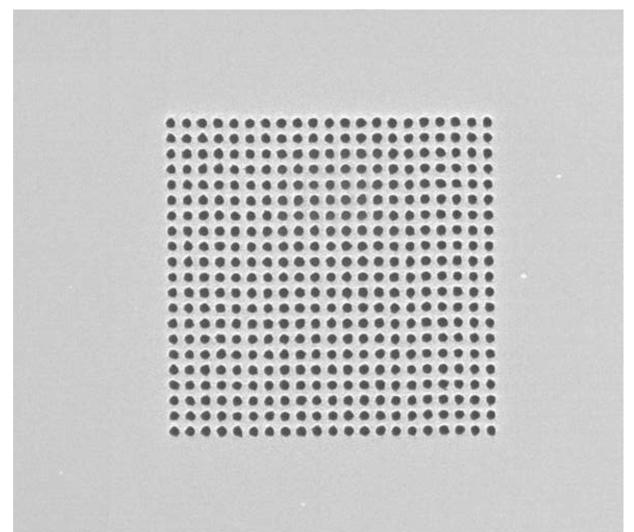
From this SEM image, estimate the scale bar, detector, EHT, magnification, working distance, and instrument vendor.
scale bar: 10000 nm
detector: ETD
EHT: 5 kV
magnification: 3.5 K X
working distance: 4.1 mm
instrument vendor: FEI